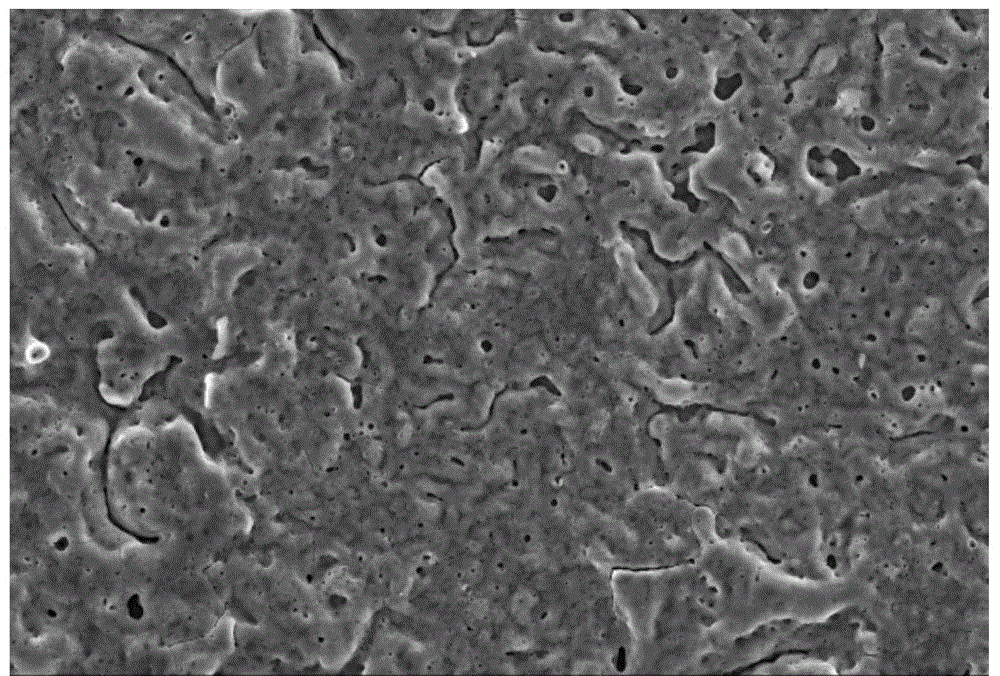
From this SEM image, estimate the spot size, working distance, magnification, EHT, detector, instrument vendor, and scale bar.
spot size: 30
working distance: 8 mm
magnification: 0.5 K X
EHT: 20 kV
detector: SEI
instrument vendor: JEOL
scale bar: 50000 nm